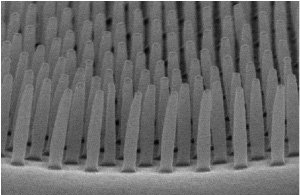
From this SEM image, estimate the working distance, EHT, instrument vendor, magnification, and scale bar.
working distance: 11.3 mm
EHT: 15 kV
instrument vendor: Hitachi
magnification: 40 K X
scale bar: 1000 nm